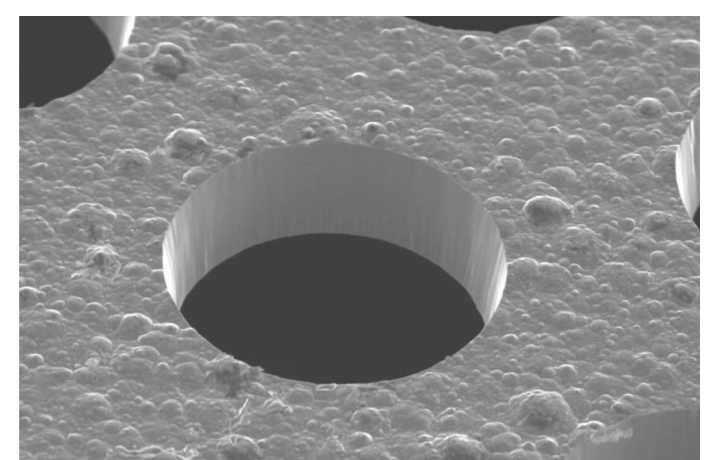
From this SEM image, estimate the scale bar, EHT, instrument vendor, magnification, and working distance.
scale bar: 20000 nm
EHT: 20 kV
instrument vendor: Hitachi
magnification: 0.5 K X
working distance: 20.5 mm